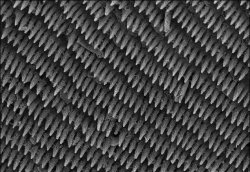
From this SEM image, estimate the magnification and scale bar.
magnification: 0.5 K X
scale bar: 50000 nm